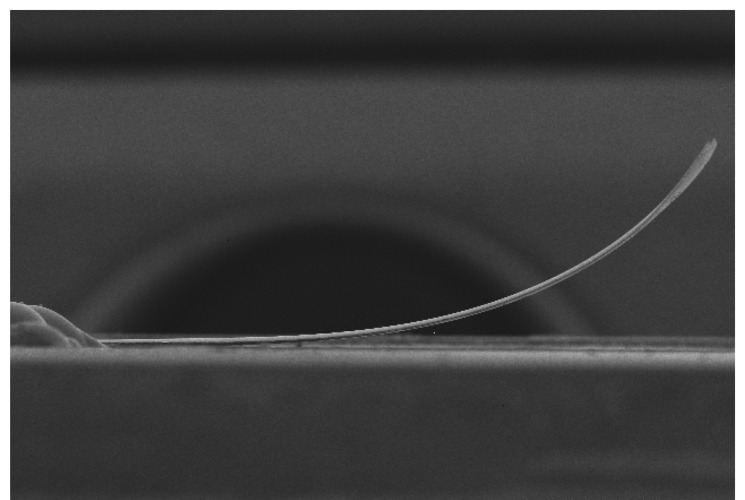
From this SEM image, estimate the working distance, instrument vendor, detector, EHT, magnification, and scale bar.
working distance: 19.8 mm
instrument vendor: Hitachi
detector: SE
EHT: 10 kV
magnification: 0.035 K X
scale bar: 1000000 nm